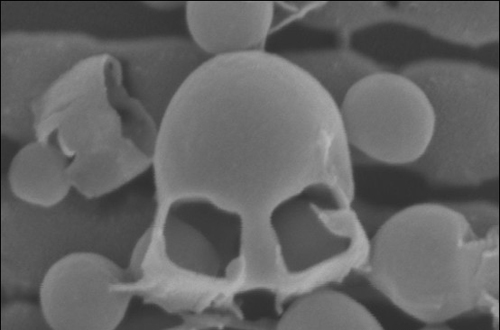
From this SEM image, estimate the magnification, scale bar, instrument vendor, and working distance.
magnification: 424.28 K X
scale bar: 100 nm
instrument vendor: Zeiss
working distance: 6 mm